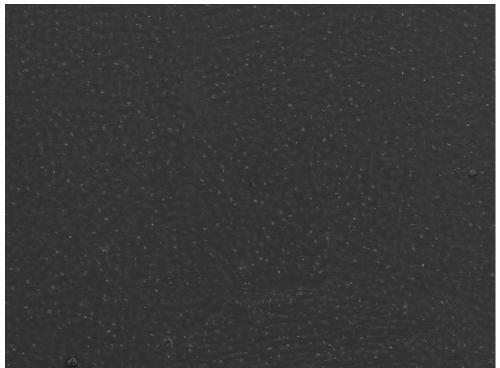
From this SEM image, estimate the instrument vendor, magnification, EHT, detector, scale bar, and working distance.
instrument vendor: JEOL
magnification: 0.2 K X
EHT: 5 kV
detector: LED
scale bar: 100000 nm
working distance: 7.8 mm